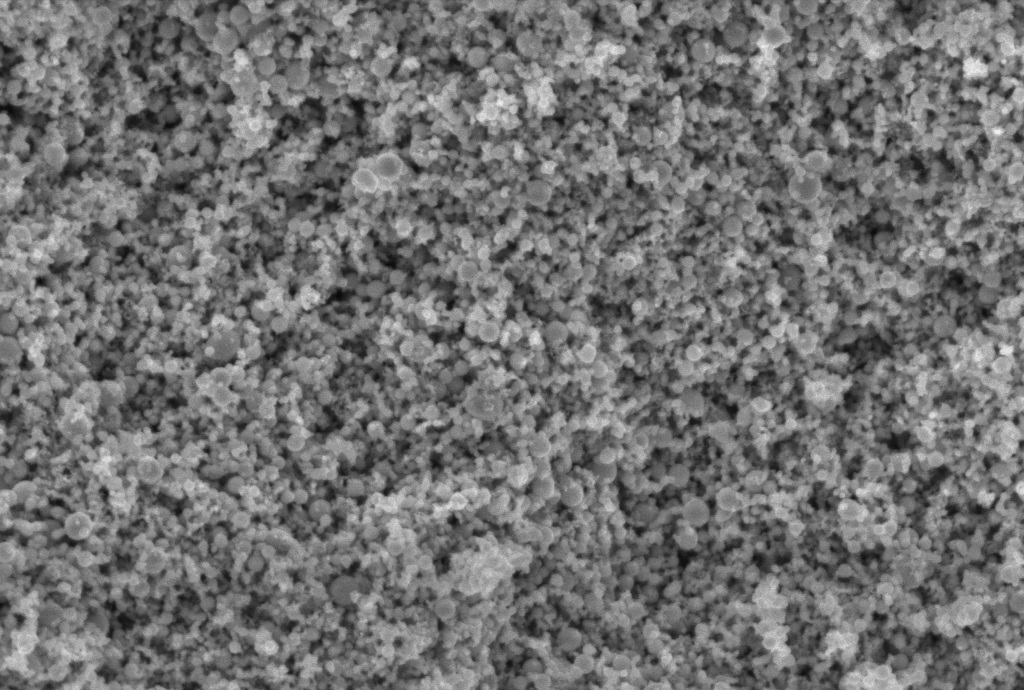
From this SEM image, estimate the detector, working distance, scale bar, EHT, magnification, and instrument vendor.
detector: InLens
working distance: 6.3 mm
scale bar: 200 nm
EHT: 5 kV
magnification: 30 K X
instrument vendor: Zeiss